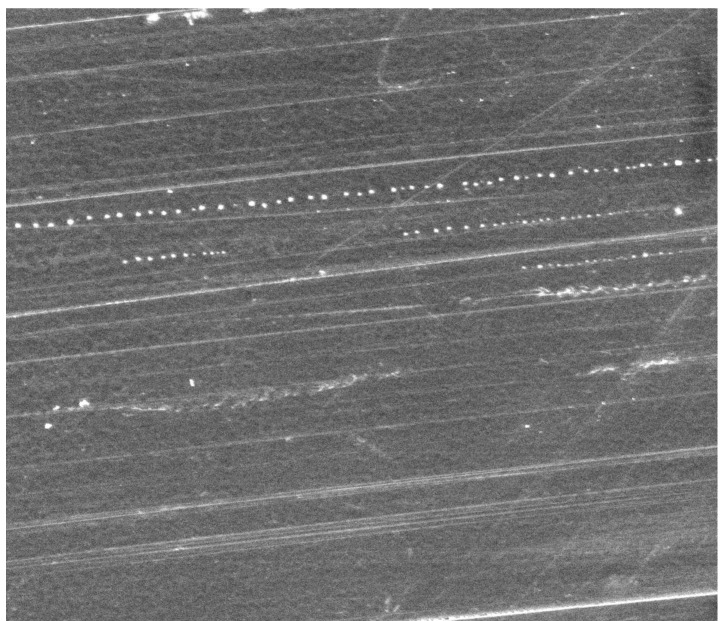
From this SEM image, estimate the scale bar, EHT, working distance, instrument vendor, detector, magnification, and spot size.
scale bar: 100000 nm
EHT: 15 kV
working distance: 24.9 mm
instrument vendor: FEI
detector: ETD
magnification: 1 K X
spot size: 6